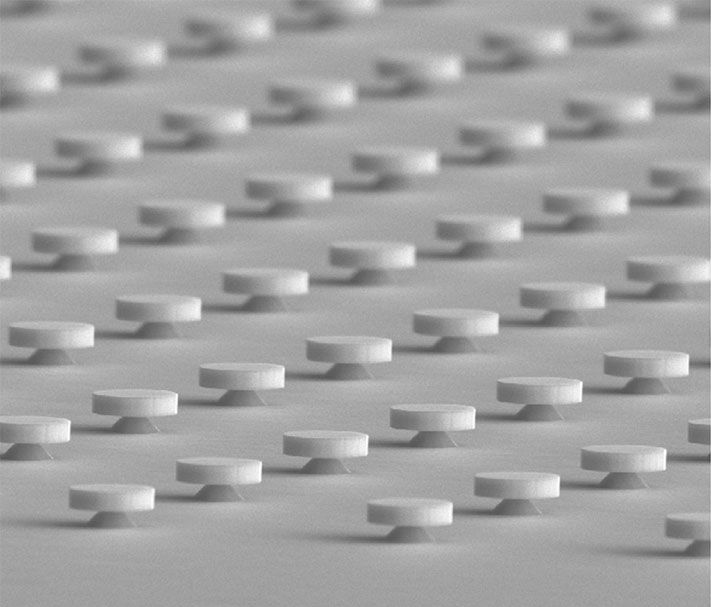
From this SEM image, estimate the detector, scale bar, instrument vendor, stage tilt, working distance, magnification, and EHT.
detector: ETD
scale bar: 10000 nm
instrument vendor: FEI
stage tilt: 44°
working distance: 6.7 mm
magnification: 6 K X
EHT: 10 kV